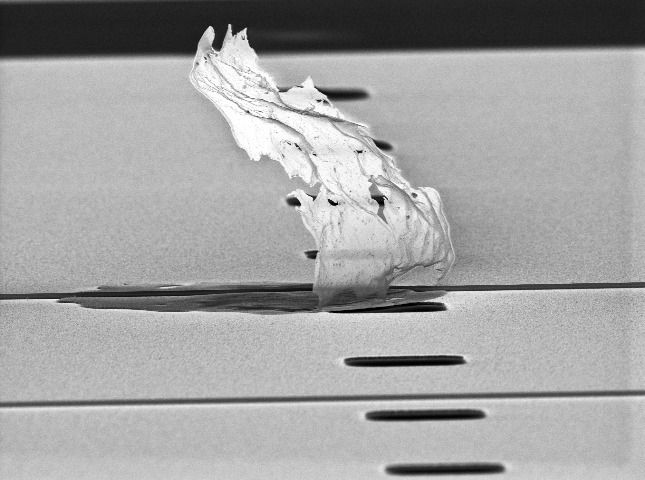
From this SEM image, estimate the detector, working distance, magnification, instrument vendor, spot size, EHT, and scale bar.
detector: SE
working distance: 18.4 mm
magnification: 2.394 K X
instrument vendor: FEI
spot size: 3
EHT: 10 kV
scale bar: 10000 nm